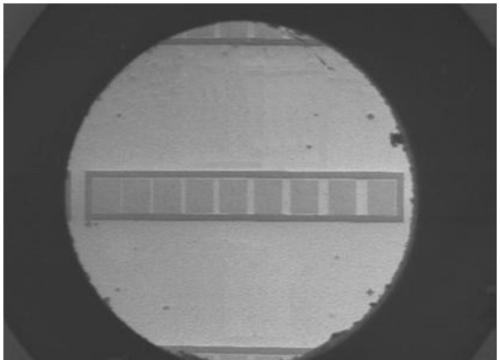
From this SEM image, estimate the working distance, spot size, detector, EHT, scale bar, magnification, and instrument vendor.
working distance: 5.2 mm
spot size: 3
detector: SE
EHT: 5 kV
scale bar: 500000 nm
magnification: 0.036 K X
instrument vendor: FEI